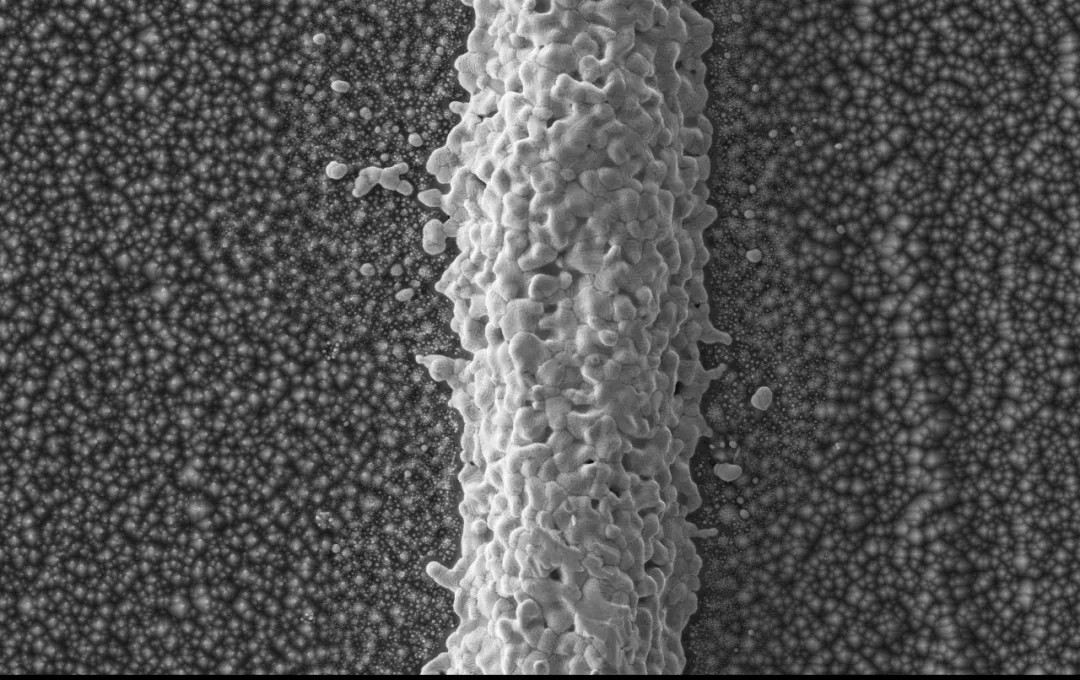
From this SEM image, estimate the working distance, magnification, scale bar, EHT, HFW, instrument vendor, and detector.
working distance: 4.829 mm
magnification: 1 K X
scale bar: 30000 nm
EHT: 15 kV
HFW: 127 µm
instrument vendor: other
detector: SED